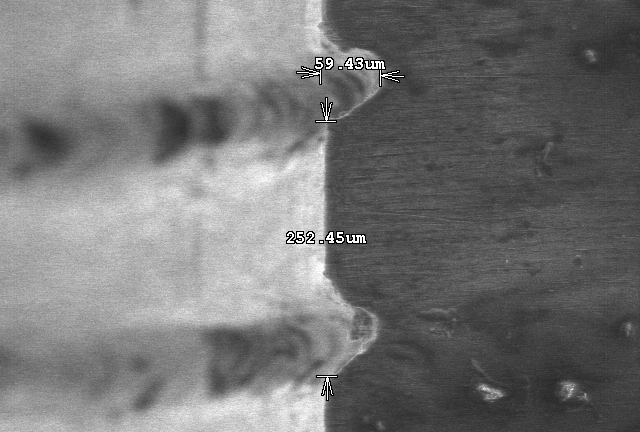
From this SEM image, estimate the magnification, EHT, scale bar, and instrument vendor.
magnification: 0.2 K X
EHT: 15 kV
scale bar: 200000 nm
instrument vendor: Hitachi